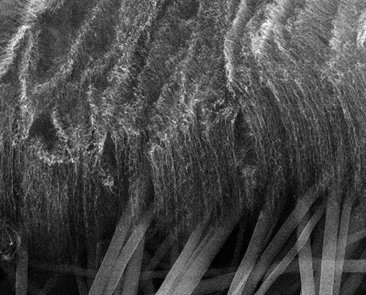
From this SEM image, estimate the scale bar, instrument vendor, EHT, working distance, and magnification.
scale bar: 3000 nm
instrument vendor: FEI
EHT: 15 kV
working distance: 16 mm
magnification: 8 K X